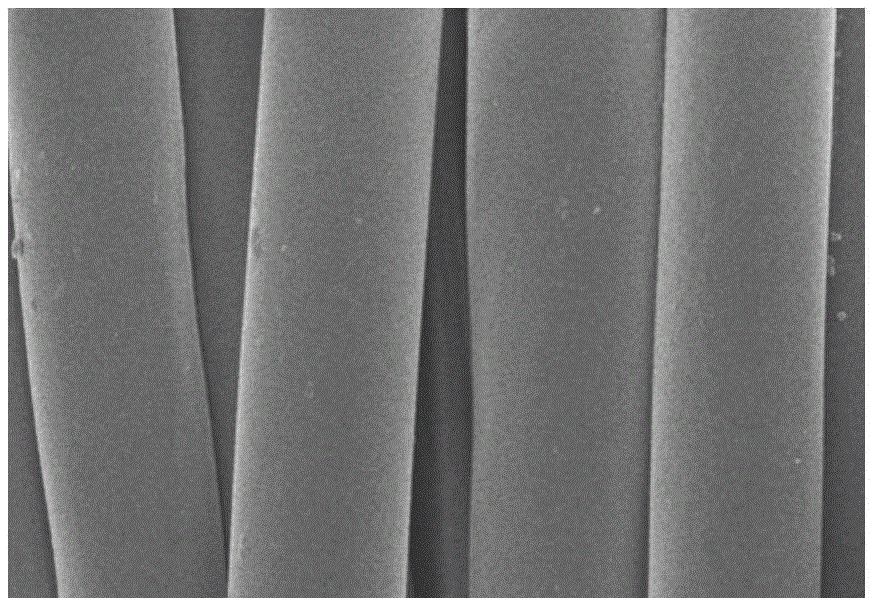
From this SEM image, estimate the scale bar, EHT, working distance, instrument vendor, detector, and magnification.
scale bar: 20000 nm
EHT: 5 kV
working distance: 10.7 mm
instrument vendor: Hitachi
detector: SE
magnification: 2 K X